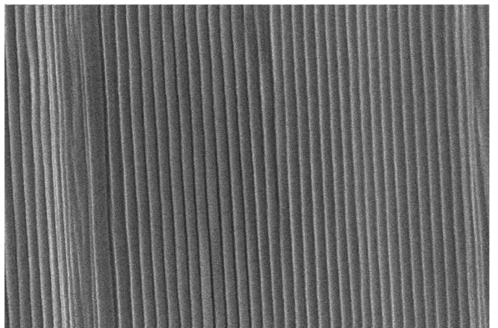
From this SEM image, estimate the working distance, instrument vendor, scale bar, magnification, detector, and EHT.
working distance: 2.5 mm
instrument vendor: Zeiss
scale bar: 200 nm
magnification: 30 K X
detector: InLens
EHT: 8 kV